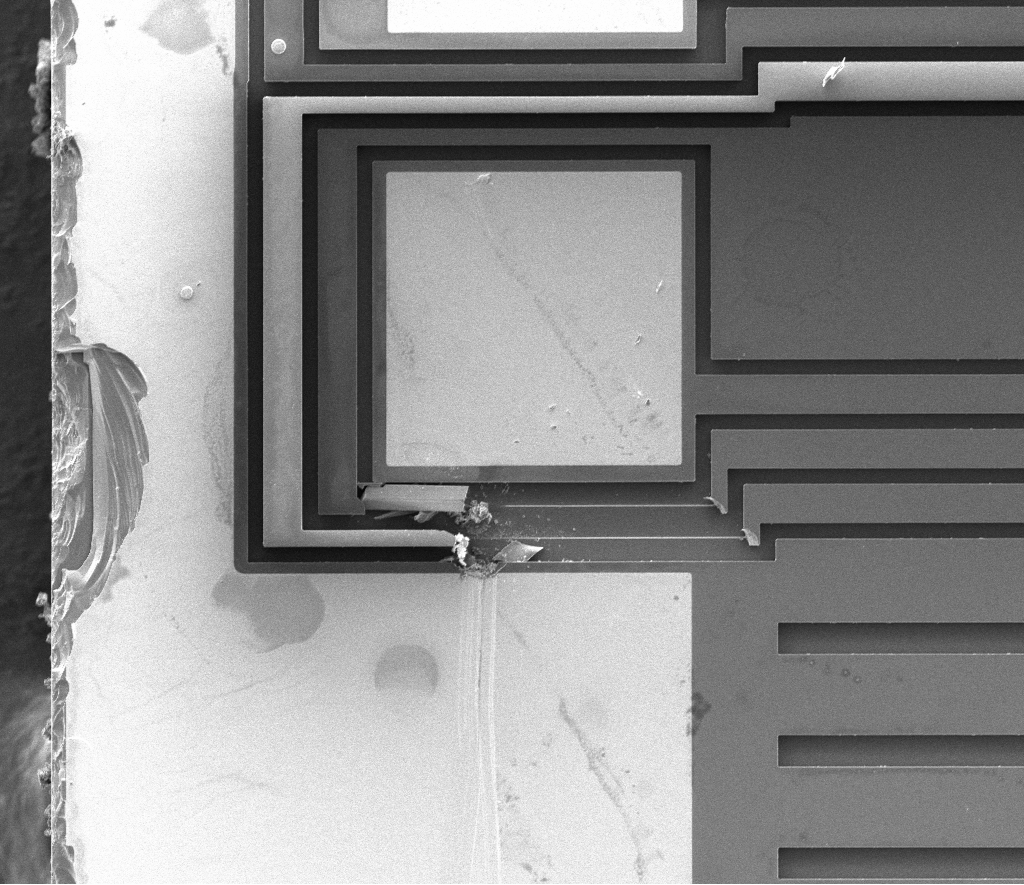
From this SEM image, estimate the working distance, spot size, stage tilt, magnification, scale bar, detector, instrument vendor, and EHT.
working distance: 10.3 mm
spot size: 3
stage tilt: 0°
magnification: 0.4 K X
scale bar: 200000 nm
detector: ETD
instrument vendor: FEI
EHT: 5 kV